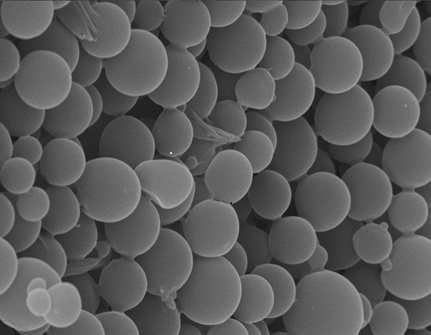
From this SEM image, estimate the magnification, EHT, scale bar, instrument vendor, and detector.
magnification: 20 K X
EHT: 20 kV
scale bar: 1000 nm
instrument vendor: Zeiss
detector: SE1